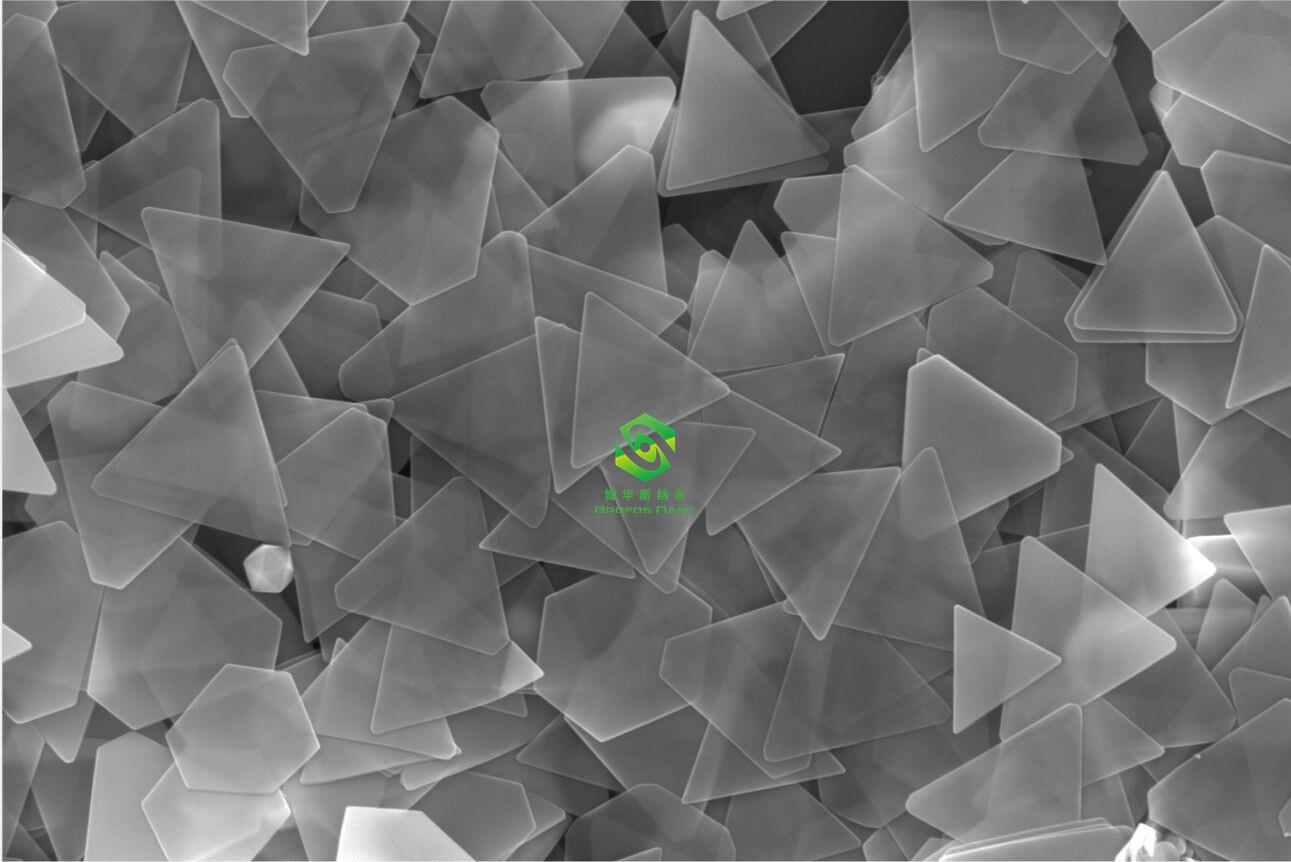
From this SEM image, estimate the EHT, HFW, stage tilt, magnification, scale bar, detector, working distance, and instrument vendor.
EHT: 10 kV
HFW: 5.18 µm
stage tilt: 0°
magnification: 80 K X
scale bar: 1000 nm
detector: TLD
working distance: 4 mm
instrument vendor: FEI